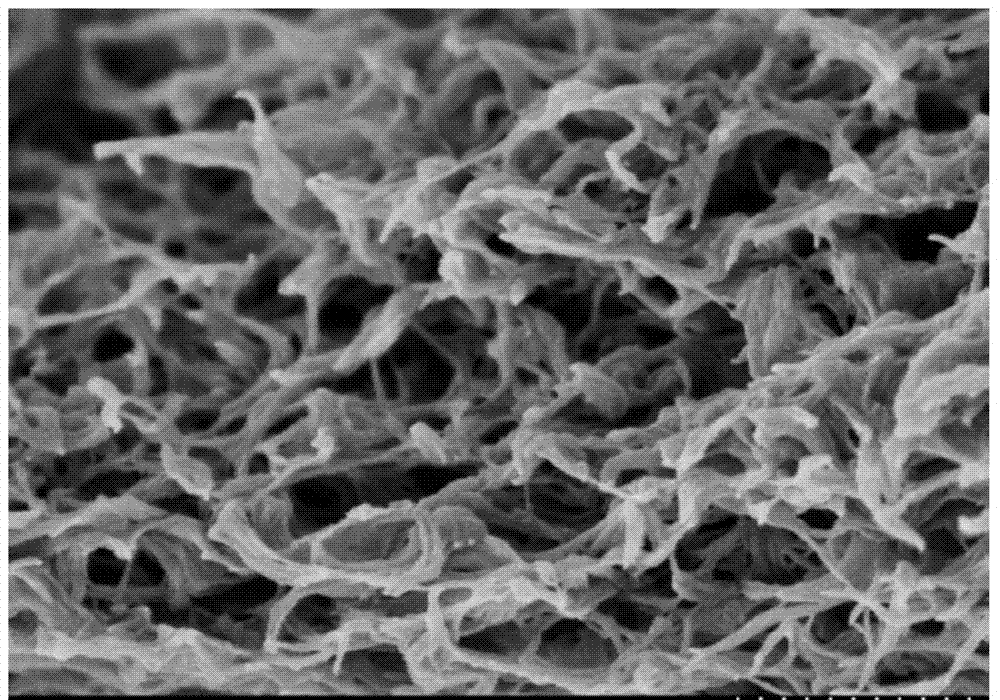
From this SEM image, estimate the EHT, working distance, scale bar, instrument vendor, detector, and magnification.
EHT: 2 kV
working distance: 4.1 mm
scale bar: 500 nm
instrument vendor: Hitachi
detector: SE(M)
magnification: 60 K X